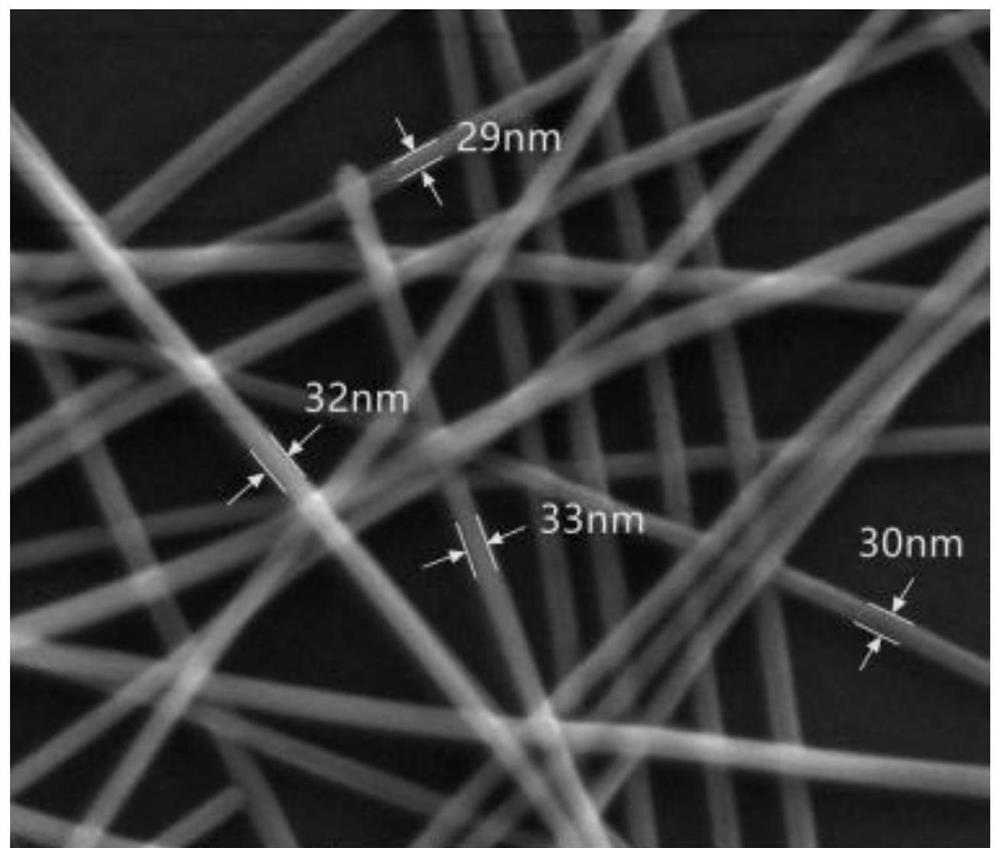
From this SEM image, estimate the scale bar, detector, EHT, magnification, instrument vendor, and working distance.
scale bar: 400 nm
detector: SE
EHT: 20 kV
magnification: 200 K X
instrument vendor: FEI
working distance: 6.4 mm